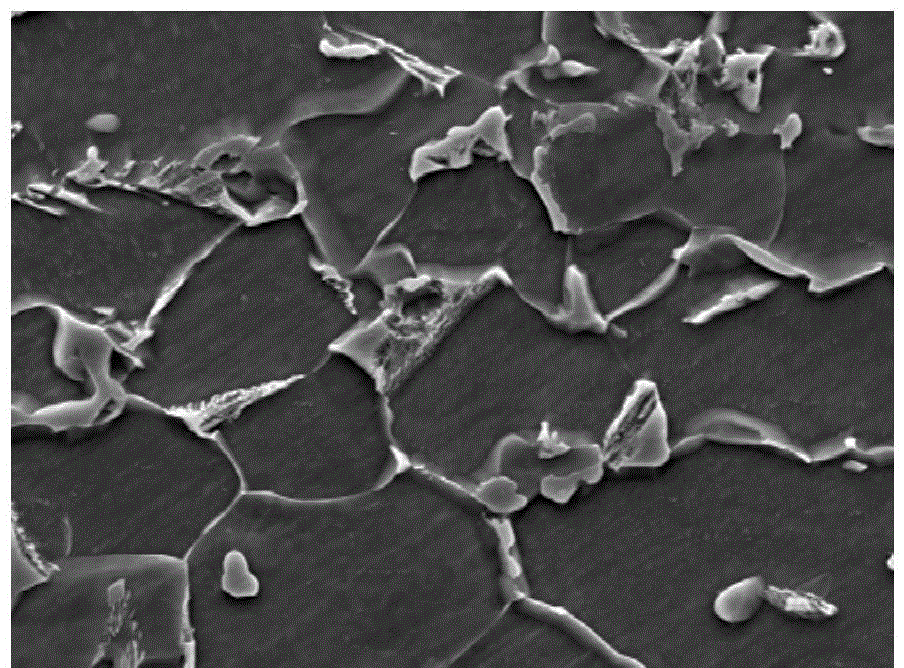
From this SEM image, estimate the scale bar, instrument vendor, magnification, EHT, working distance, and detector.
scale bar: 1000 nm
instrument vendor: JEOL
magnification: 5 K X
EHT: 15 kV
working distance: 9 mm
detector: SEI